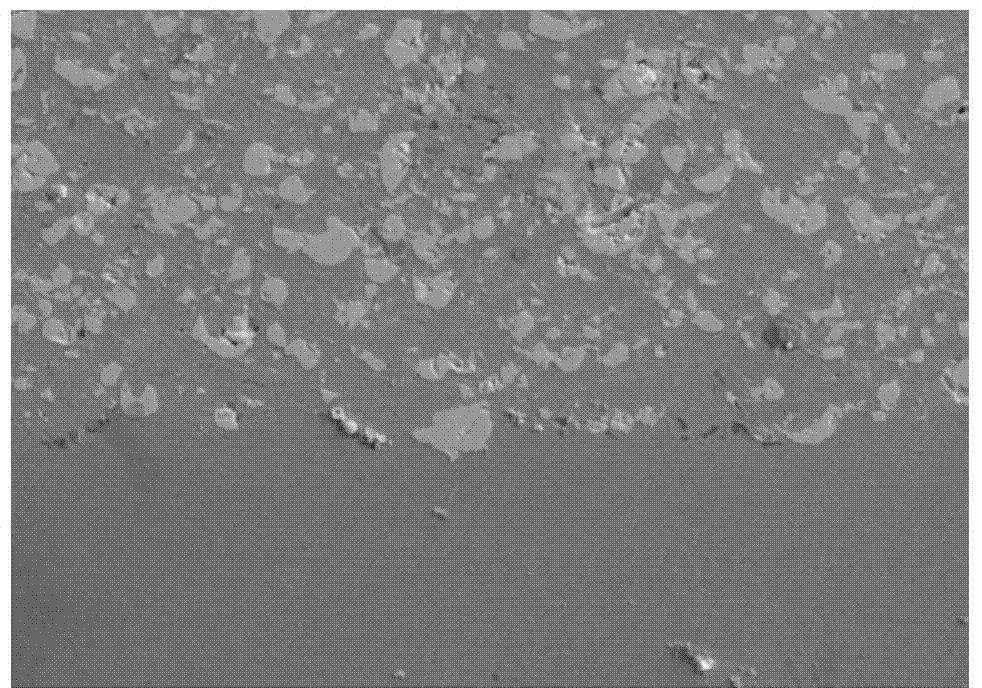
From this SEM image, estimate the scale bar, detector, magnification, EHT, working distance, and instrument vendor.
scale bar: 20000 nm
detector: SE2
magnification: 0.2 K X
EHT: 15 kV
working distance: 8.2 mm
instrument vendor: Zeiss